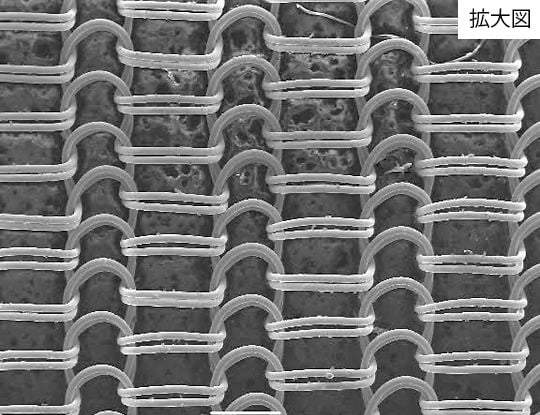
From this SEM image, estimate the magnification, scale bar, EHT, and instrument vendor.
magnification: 0.03 K X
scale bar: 500000 nm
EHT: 20 kV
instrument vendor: JEOL